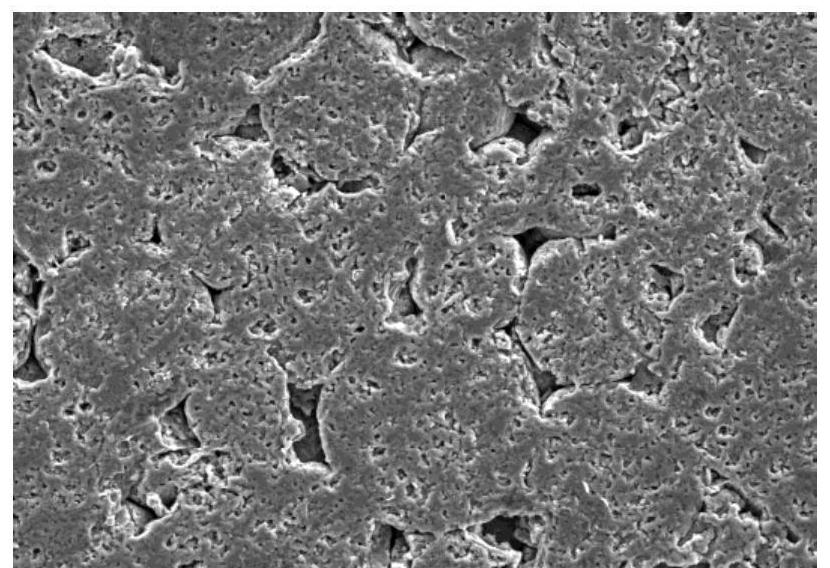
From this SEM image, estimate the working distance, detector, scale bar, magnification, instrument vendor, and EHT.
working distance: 8.4 mm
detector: LM(UL)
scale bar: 100000 nm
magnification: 0.35 K X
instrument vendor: Hitachi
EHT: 20 kV